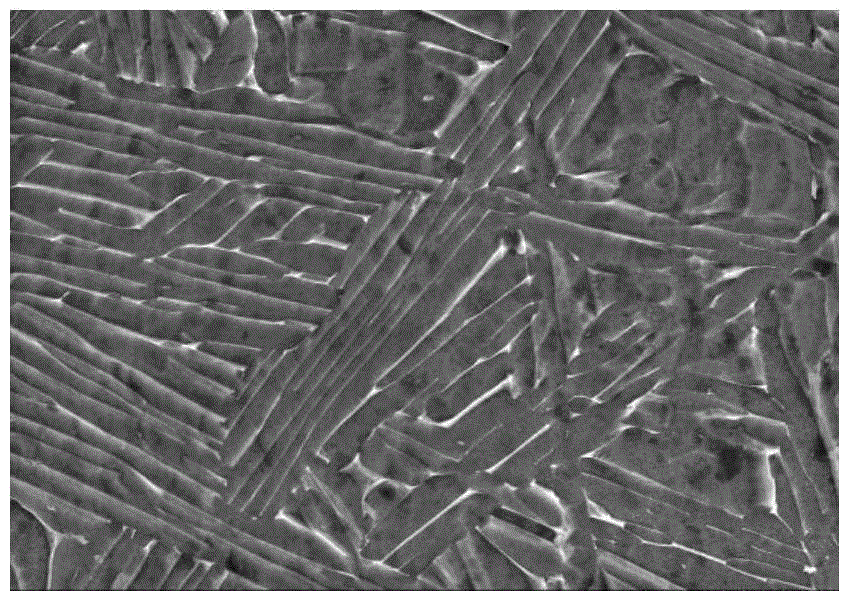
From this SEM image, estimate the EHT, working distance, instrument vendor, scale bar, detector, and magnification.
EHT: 15 kV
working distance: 13.8 mm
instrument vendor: Hitachi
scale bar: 10000 nm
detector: SE(M)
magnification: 3 K X